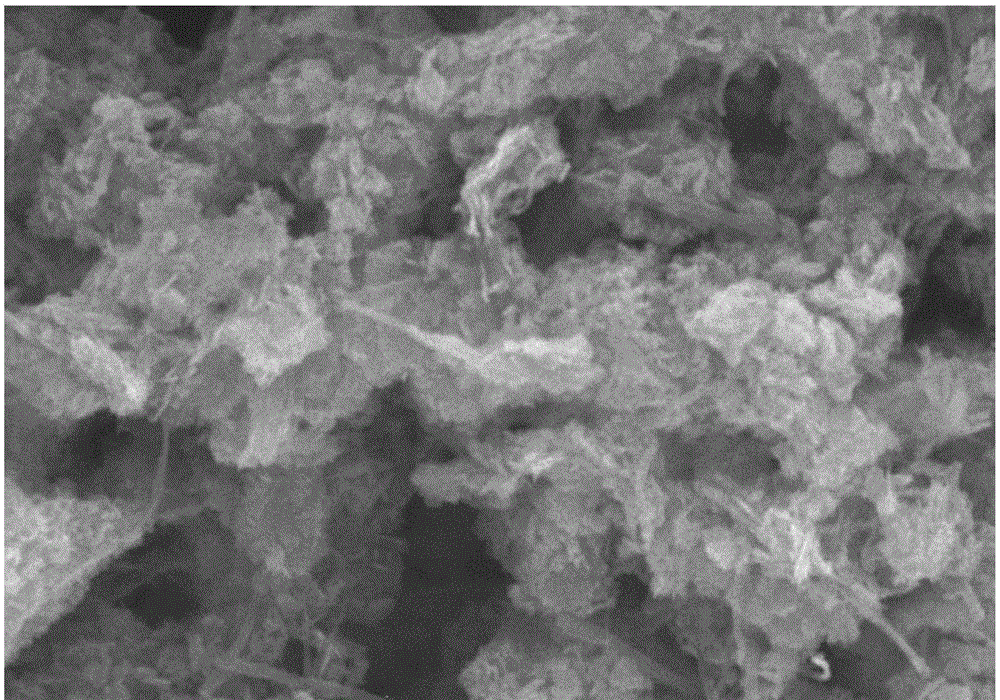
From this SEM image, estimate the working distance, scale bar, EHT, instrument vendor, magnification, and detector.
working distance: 9.6 mm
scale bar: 5000 nm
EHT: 15 kV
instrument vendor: Hitachi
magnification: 9.99 K X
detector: SE